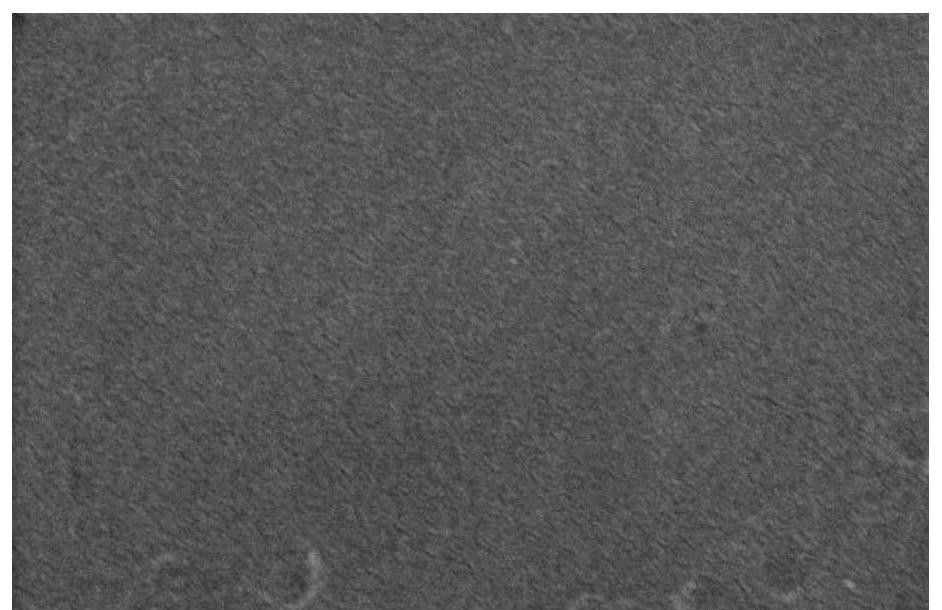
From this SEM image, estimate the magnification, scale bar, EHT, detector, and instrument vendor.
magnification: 30 K X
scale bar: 1000 nm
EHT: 10 kV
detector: SE(M)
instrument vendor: Hitachi